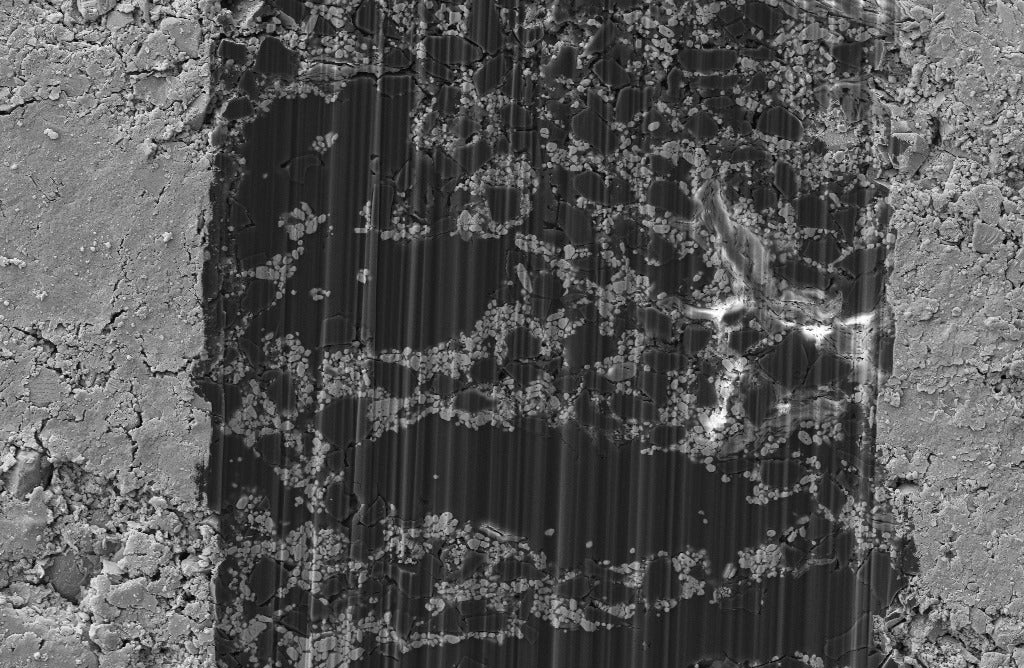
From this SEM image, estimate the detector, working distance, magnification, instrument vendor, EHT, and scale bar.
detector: SE2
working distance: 7.1 mm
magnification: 2 K X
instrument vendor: Zeiss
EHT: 5 kV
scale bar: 2000 nm